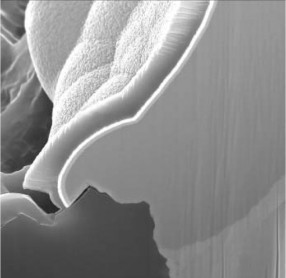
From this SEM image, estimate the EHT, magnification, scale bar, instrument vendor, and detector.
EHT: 10 kV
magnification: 11 K X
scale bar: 2000 nm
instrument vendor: other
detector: SEM